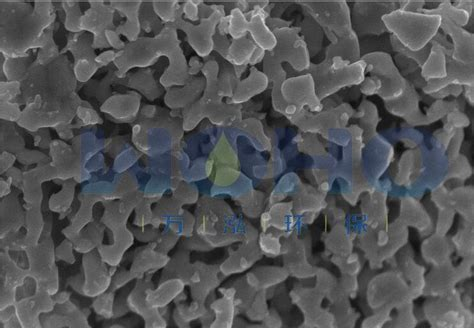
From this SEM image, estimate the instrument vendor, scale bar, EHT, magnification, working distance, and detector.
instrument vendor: Hitachi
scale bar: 1000 nm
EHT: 5 kV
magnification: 50 K X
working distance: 2.1 mm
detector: SE(U)